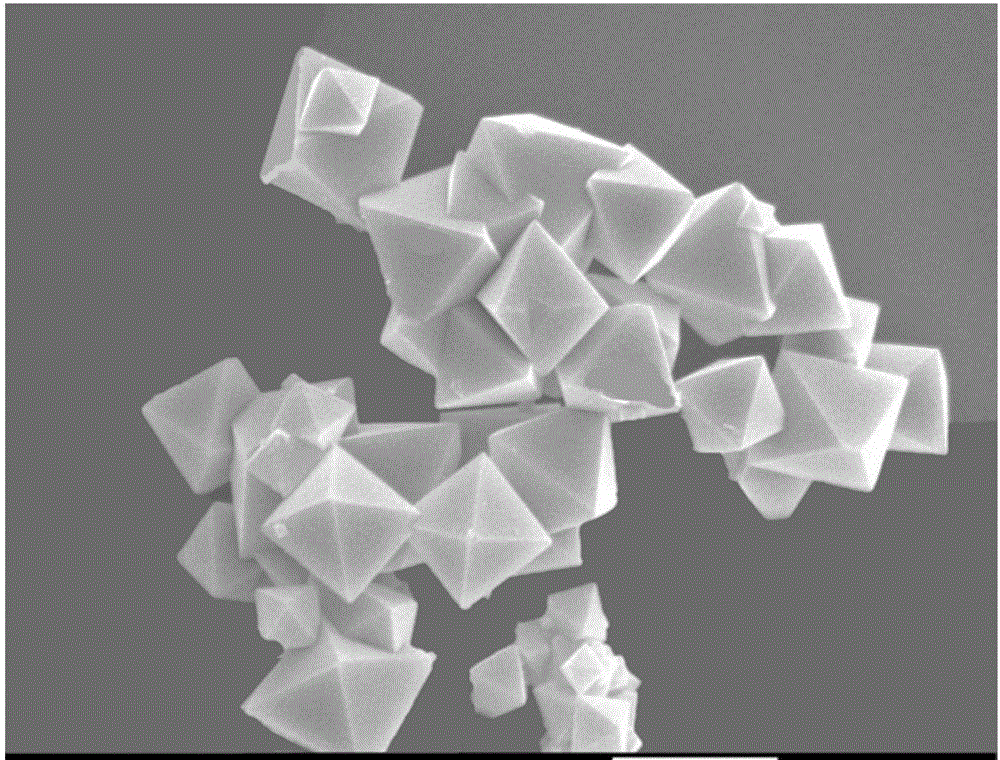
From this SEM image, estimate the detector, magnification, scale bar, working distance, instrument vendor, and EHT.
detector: SEI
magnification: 20 K X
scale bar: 1000 nm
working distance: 7.7 mm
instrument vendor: JEOL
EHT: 8 kV